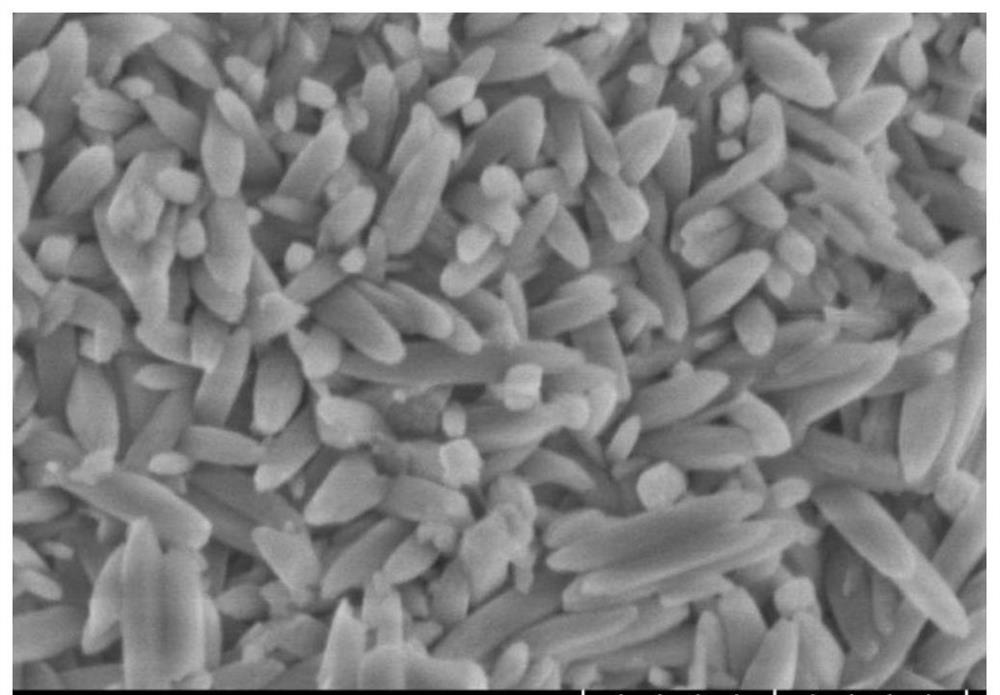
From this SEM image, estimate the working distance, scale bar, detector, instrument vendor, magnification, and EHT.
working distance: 9.4 mm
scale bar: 1000 nm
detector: SE(UL)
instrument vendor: Hitachi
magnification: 50 K X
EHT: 3 kV